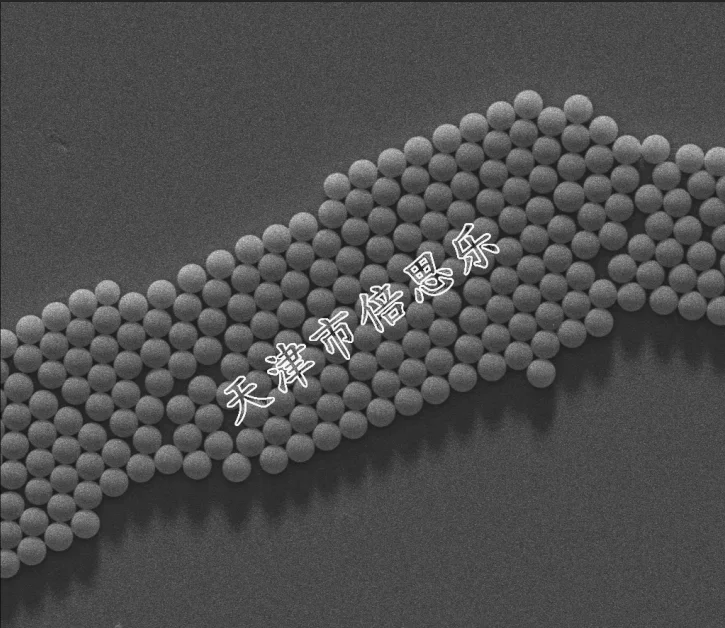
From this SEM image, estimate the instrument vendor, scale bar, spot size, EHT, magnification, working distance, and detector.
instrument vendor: FEI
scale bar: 10000 nm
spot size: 4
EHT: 10 kV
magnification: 5 K X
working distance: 8.9 mm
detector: ETD